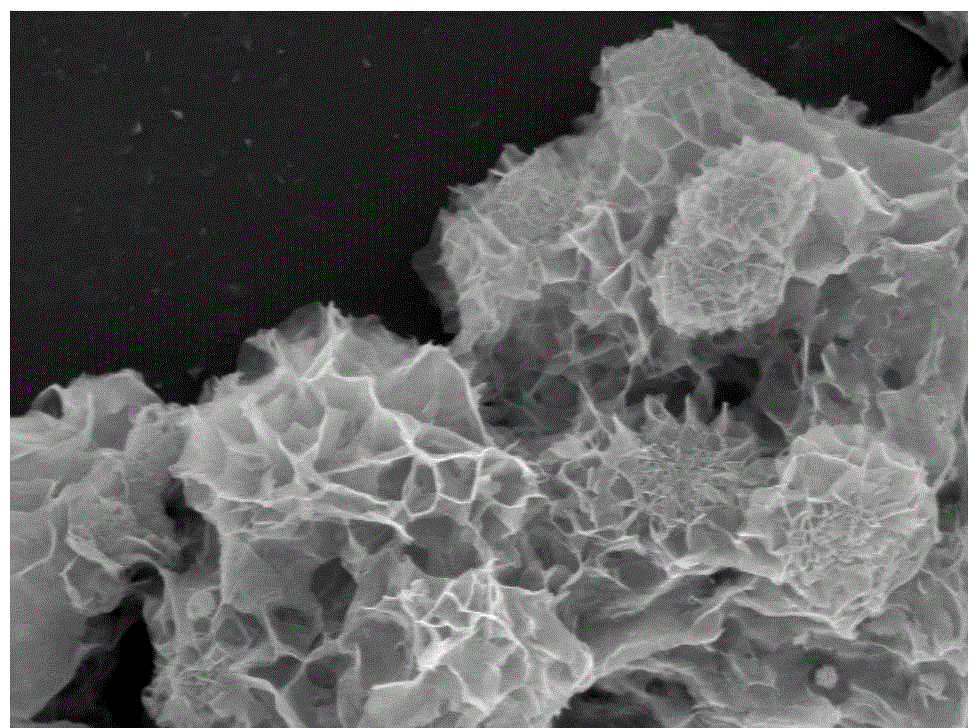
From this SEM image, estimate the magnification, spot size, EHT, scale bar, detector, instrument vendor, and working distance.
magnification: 10 K X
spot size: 5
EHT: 10 kV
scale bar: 2000 nm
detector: TLD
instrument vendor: FEI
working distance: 5 mm